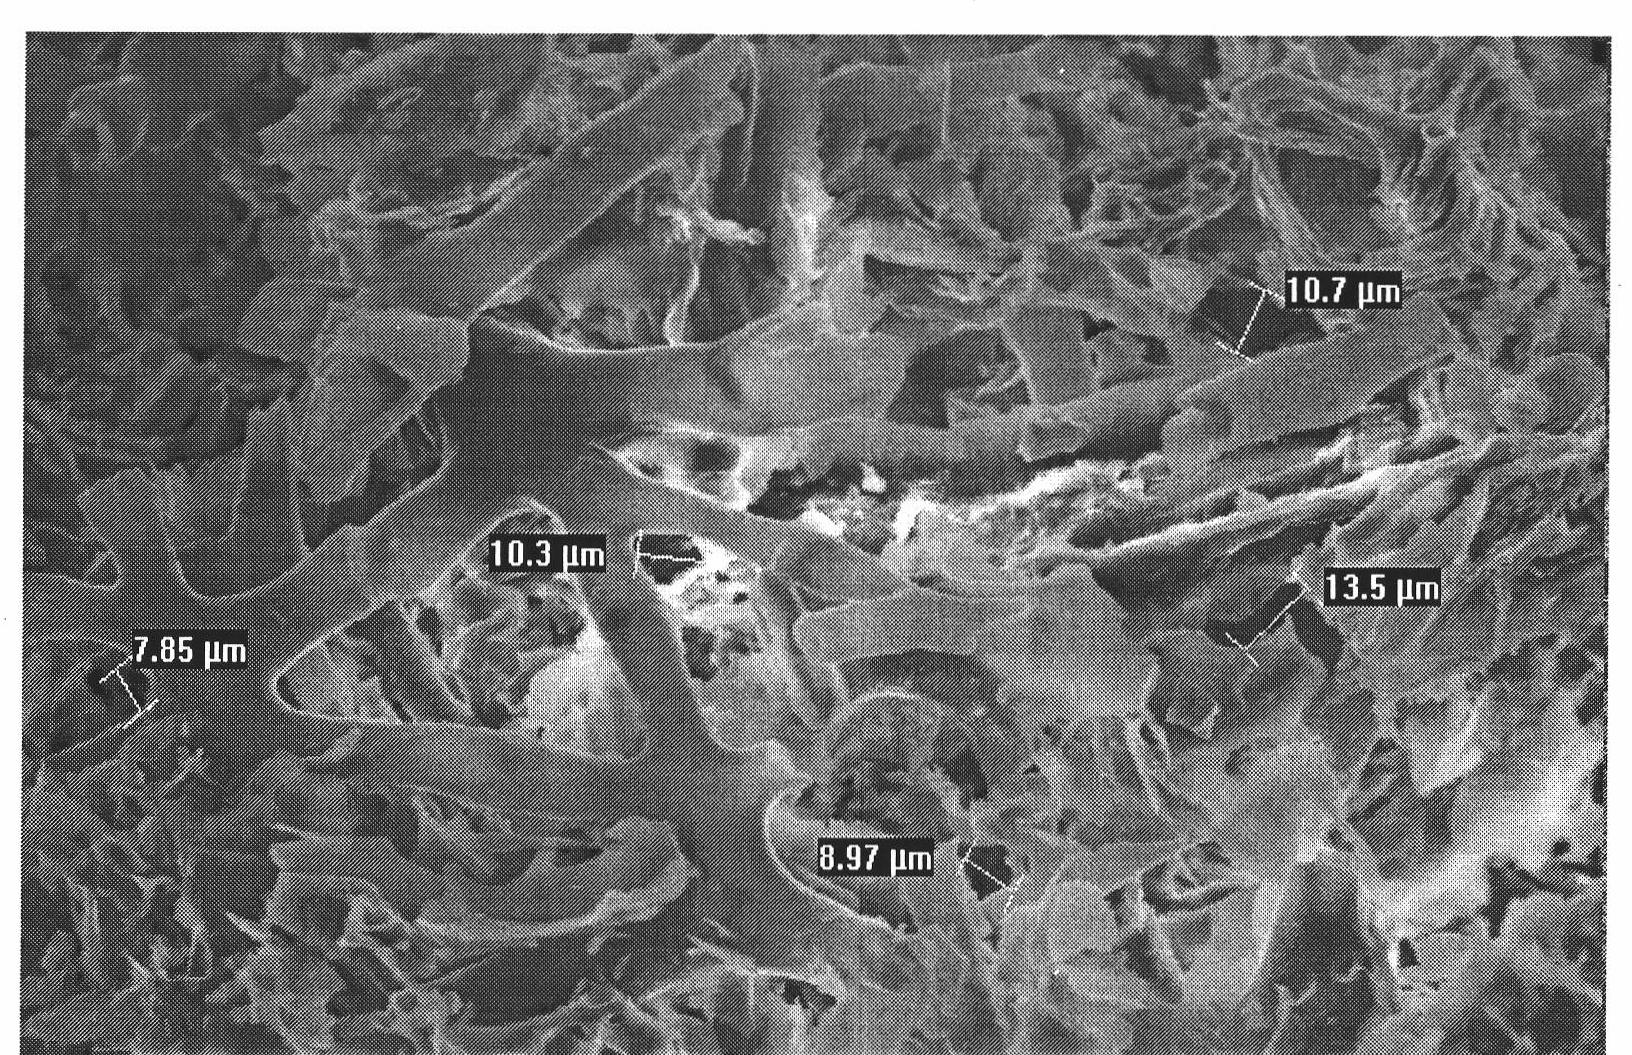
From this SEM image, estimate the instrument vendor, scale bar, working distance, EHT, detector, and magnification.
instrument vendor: FEI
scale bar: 50000 nm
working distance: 13.2 mm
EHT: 20 kV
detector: GSE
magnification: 1 K X